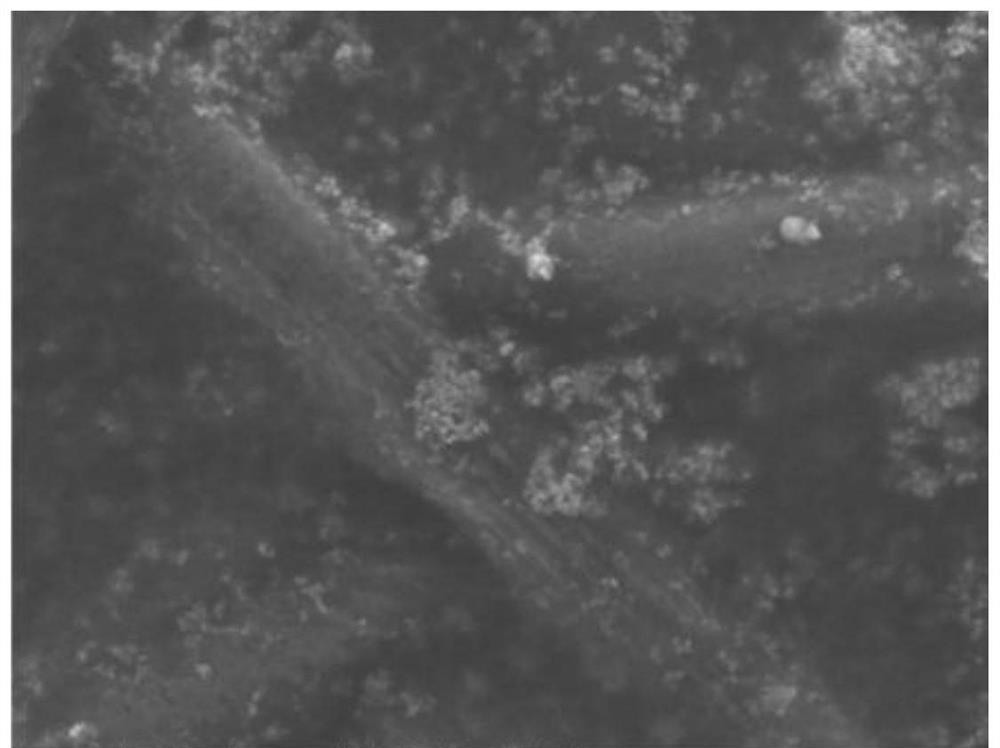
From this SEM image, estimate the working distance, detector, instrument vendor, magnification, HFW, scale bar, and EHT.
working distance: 10.1 mm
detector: ETD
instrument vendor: FEI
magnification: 5 K X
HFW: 59.7 µm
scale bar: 20000 nm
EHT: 20 kV